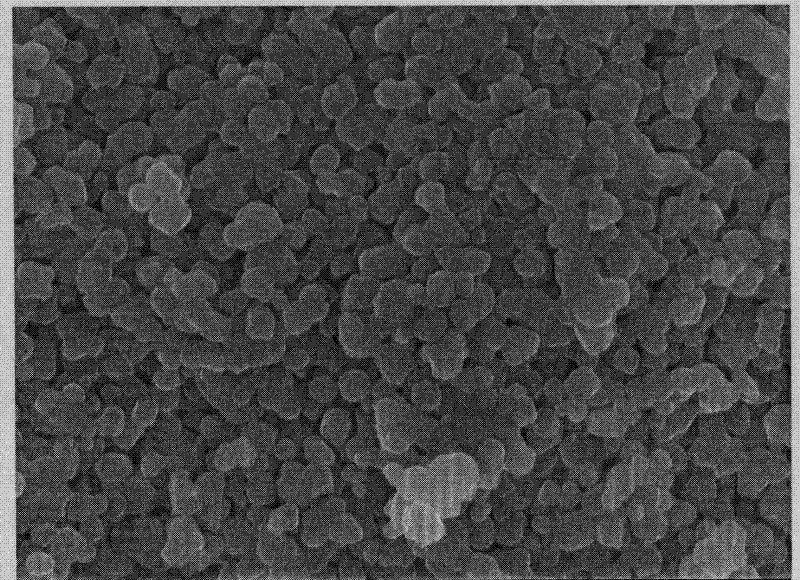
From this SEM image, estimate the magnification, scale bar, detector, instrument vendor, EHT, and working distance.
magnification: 15 K X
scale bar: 1000 nm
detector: SEI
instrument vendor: JEOL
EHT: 15 kV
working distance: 16.2 mm